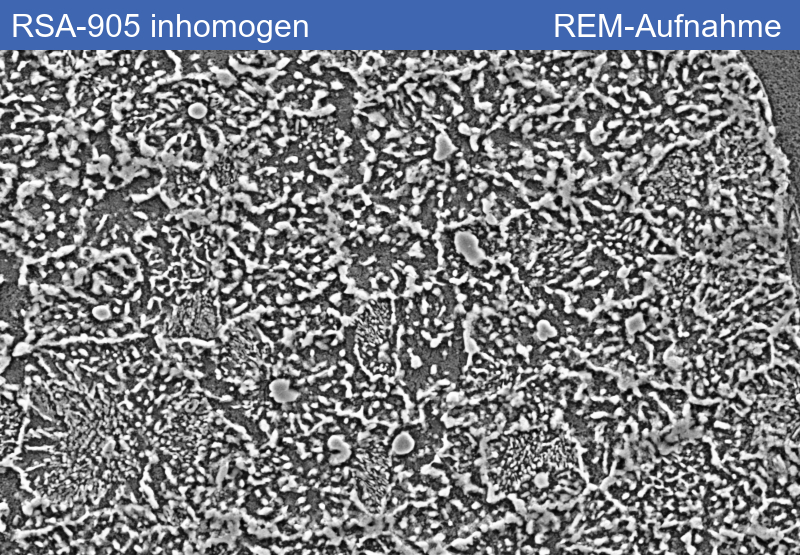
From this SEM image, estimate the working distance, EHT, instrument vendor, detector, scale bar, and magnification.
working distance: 9.4 mm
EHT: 5 kV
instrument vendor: Zeiss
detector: SE2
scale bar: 1000 nm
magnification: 5 K X